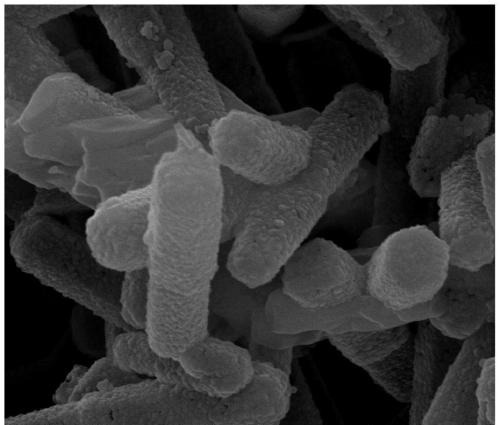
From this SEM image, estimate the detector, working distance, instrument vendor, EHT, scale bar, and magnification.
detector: SE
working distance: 10.2 mm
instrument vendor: FEI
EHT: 20 kV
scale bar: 1000 nm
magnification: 80 K X